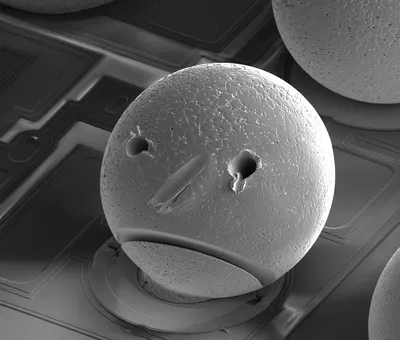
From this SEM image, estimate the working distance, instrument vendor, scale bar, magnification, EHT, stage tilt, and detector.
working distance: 4.5 mm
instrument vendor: FEI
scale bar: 200000 nm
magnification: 0.5 K X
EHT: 15 kV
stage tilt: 52°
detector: ETD